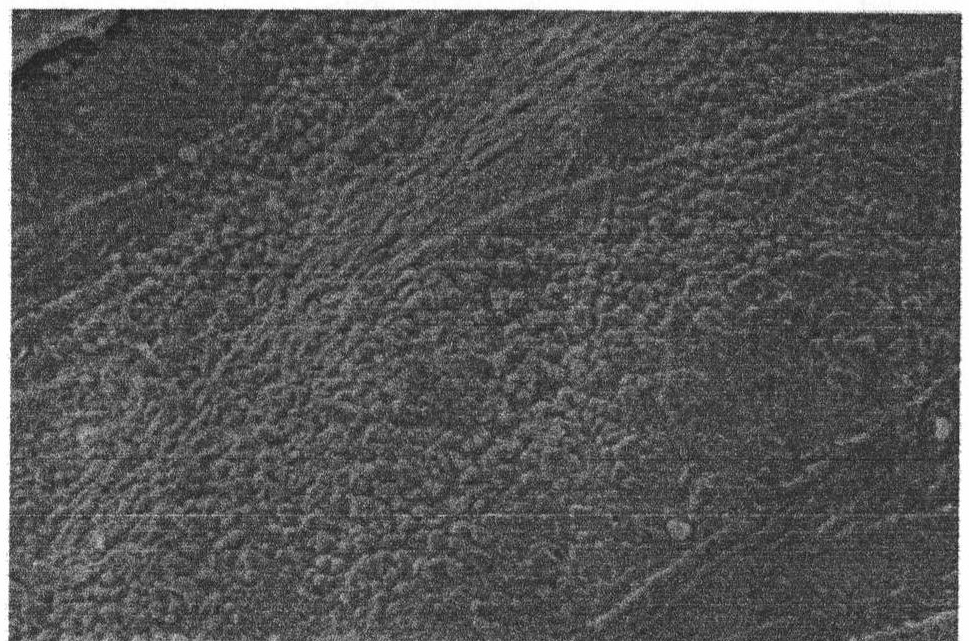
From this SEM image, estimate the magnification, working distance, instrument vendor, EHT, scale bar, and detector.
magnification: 4 K X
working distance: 12.4 mm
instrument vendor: FEI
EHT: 20 kV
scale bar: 10000 nm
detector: SE